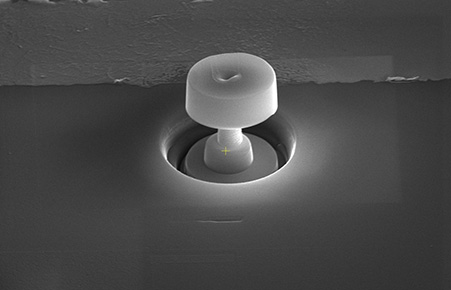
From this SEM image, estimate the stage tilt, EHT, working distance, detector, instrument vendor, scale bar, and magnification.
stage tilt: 52°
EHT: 30 kV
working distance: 4 mm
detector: ETD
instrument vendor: Thermo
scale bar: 5000 nm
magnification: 12 K X